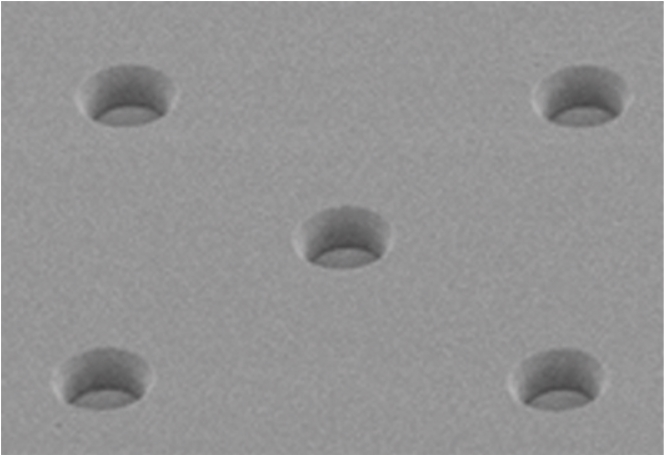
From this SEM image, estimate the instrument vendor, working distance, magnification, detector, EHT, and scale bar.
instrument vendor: Hitachi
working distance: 6.7 mm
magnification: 20 K X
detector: SE(UL)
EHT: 1 kV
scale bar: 2000 nm